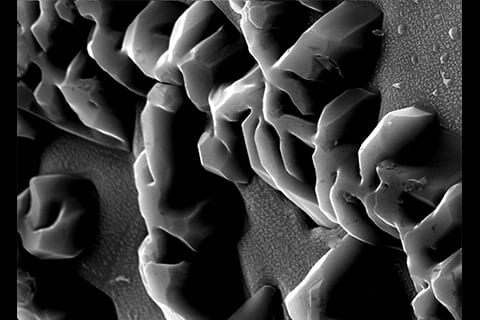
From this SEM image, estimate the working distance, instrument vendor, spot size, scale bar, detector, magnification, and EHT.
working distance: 15.5 mm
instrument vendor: FEI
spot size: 3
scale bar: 2000 nm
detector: SE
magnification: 6.383 K X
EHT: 20 kV